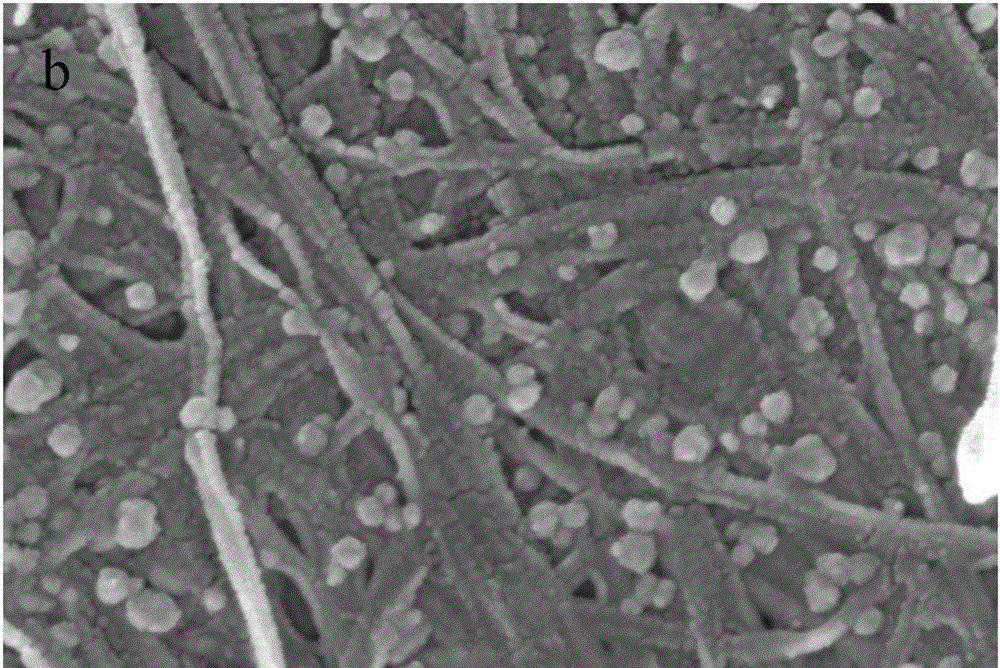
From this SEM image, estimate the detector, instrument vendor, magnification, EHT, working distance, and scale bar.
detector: InLens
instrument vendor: Zeiss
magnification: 100 K X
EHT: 5 kV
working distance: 6.8 mm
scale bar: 100 nm